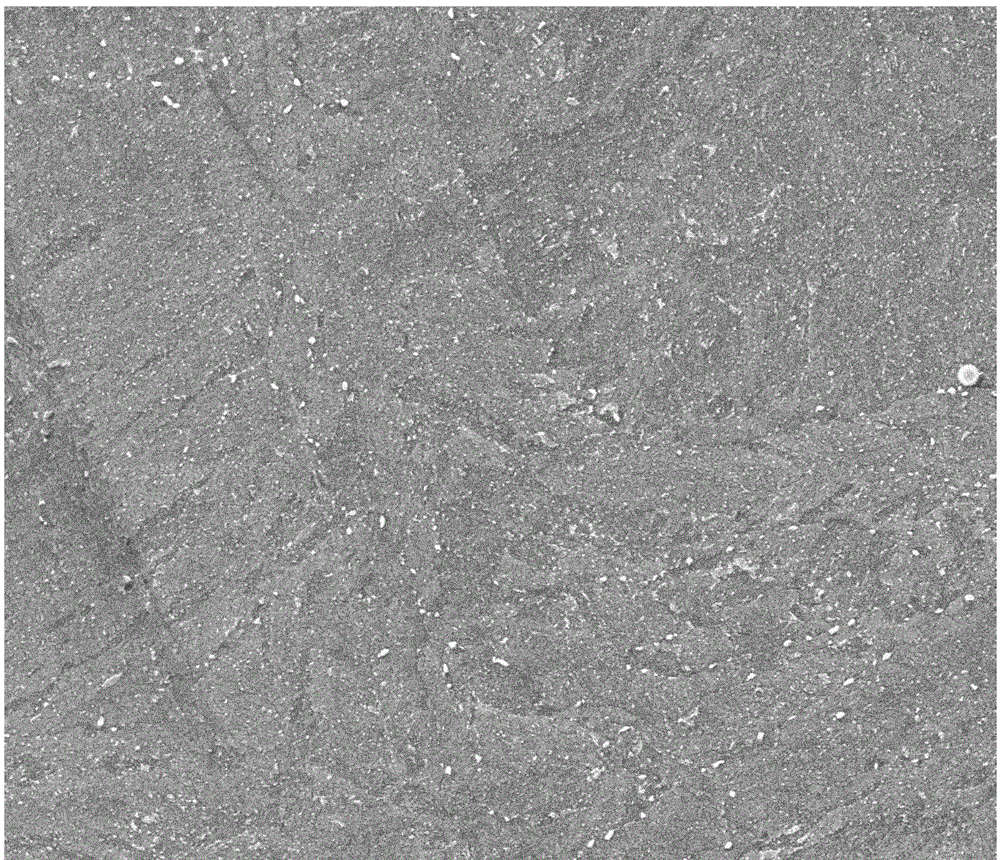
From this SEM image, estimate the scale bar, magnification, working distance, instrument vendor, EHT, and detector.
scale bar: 20000 nm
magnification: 5 K X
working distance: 9.3 mm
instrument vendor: FEI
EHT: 30 kV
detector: ETD